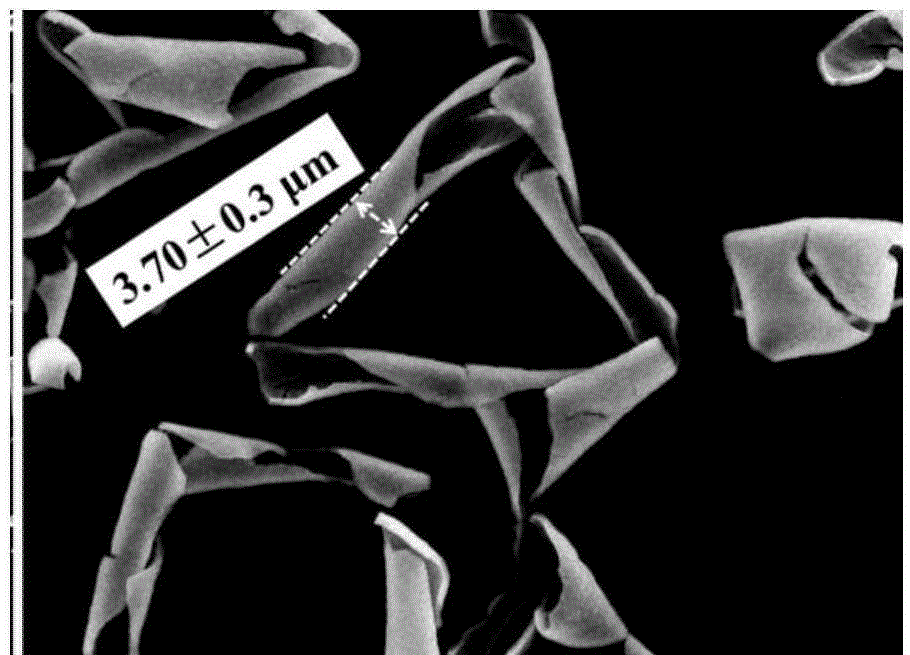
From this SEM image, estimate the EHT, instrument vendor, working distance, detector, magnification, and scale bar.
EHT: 15 kV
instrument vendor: JEOL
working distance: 8.1 mm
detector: SEI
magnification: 2 K X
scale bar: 10000 nm